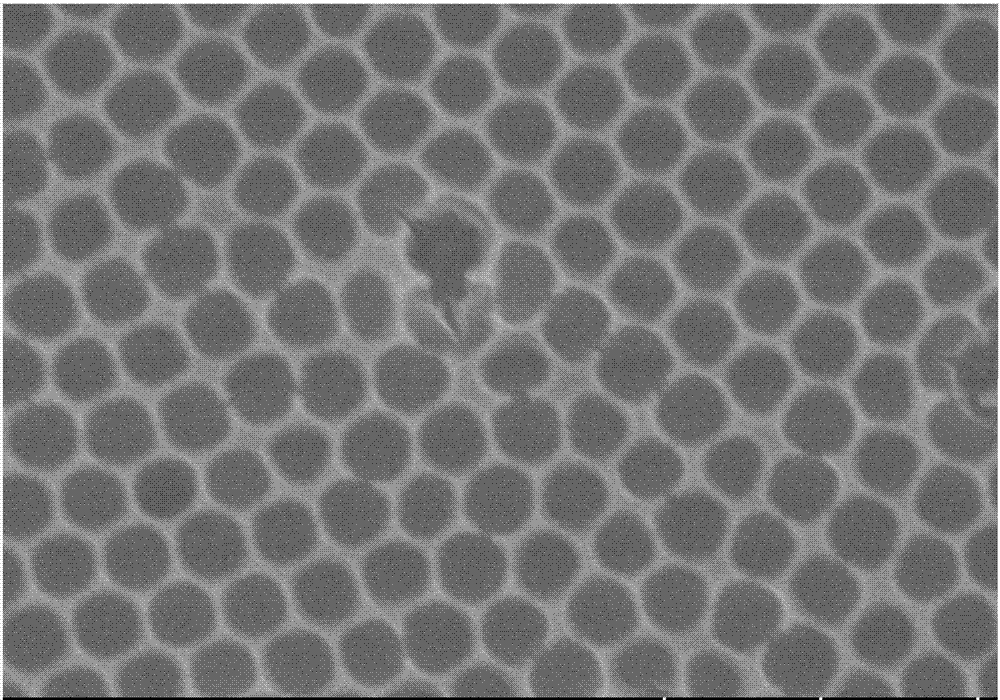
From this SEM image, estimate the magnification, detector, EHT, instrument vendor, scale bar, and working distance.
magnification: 40 K X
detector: SE(U)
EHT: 5 kV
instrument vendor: Hitachi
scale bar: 1000 nm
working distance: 9.7 mm